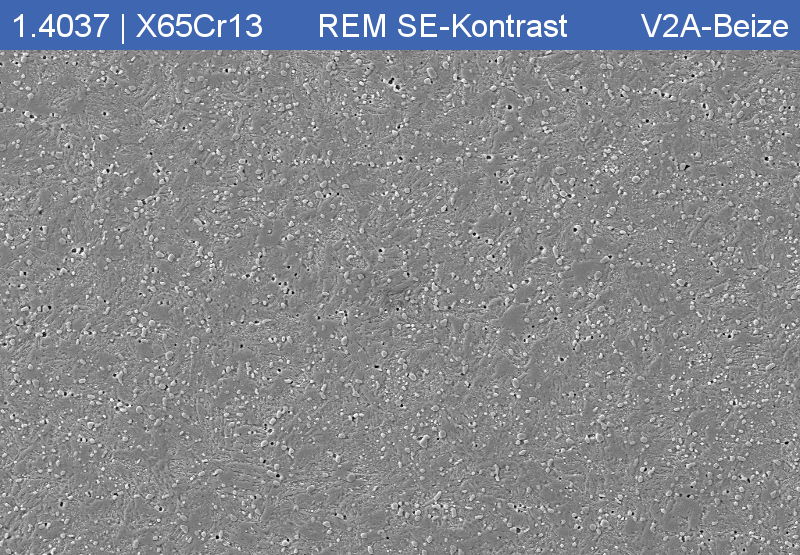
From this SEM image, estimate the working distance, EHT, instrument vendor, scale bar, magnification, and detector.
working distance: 11 mm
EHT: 15 kV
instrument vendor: Zeiss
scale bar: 10000 nm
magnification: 1 K X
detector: SE2